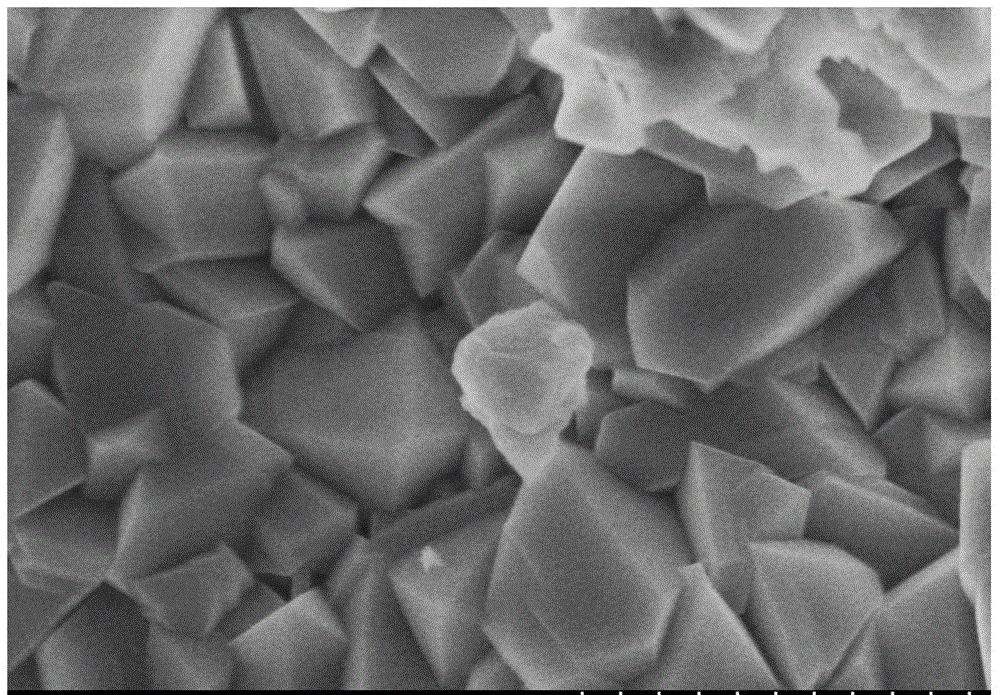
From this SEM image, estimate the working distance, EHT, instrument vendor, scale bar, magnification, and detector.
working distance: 8 mm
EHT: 3 kV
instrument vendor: Hitachi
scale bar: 1000 nm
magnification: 50 K X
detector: SE(U)